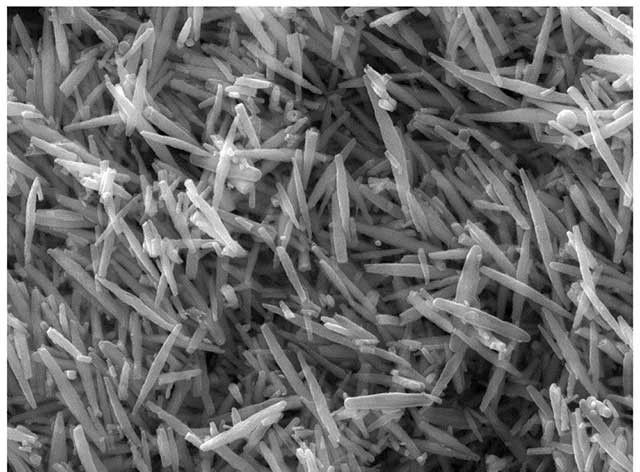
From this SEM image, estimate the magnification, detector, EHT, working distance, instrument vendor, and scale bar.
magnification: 20 K X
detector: SE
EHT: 20 kV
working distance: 15.1 mm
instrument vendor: Tescan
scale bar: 2000 nm